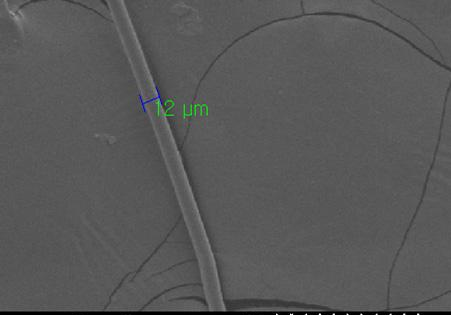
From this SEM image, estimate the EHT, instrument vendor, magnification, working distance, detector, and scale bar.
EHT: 5 kV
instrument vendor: other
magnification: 0.4 K X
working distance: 26.9 mm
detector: SE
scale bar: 100000 nm